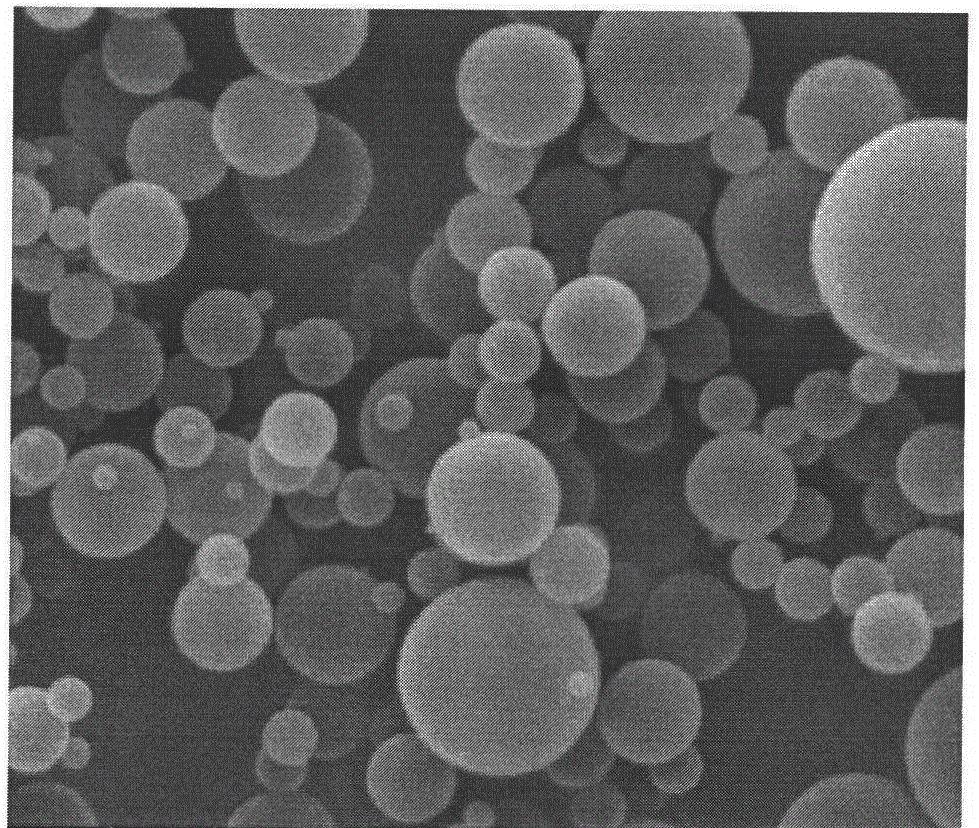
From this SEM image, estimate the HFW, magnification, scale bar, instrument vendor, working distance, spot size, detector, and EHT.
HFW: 14.9 µm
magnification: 20 K X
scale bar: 5000 nm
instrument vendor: FEI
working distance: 10.2 mm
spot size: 3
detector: ETD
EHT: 20 kV